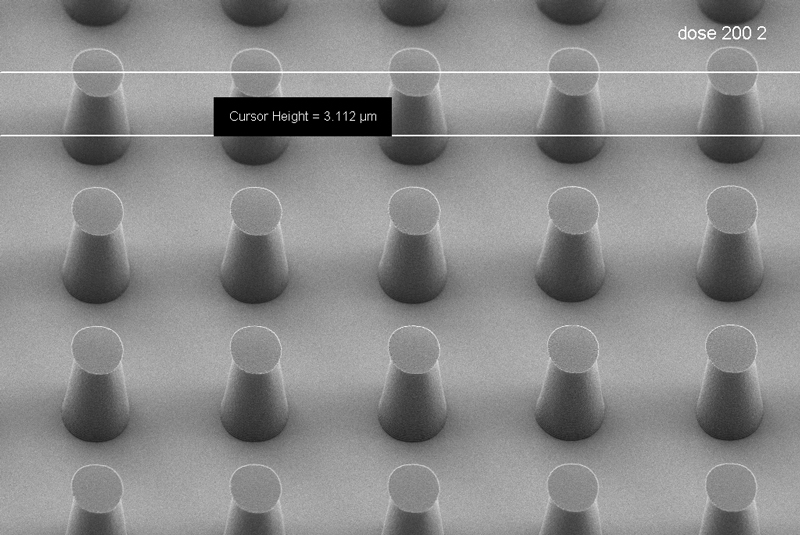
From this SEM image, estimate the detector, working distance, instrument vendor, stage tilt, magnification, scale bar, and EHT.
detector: HE-SE2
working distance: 6.6 mm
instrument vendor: Zeiss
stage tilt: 30°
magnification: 2.91 K X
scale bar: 2000 nm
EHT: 1 kV